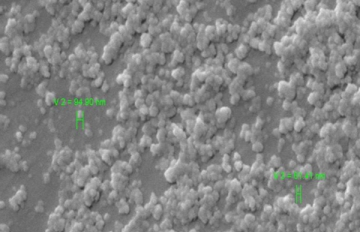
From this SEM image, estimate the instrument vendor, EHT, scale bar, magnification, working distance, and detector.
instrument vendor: Zeiss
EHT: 10 kV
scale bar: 200 nm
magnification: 20 K X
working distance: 9 mm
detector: SE1